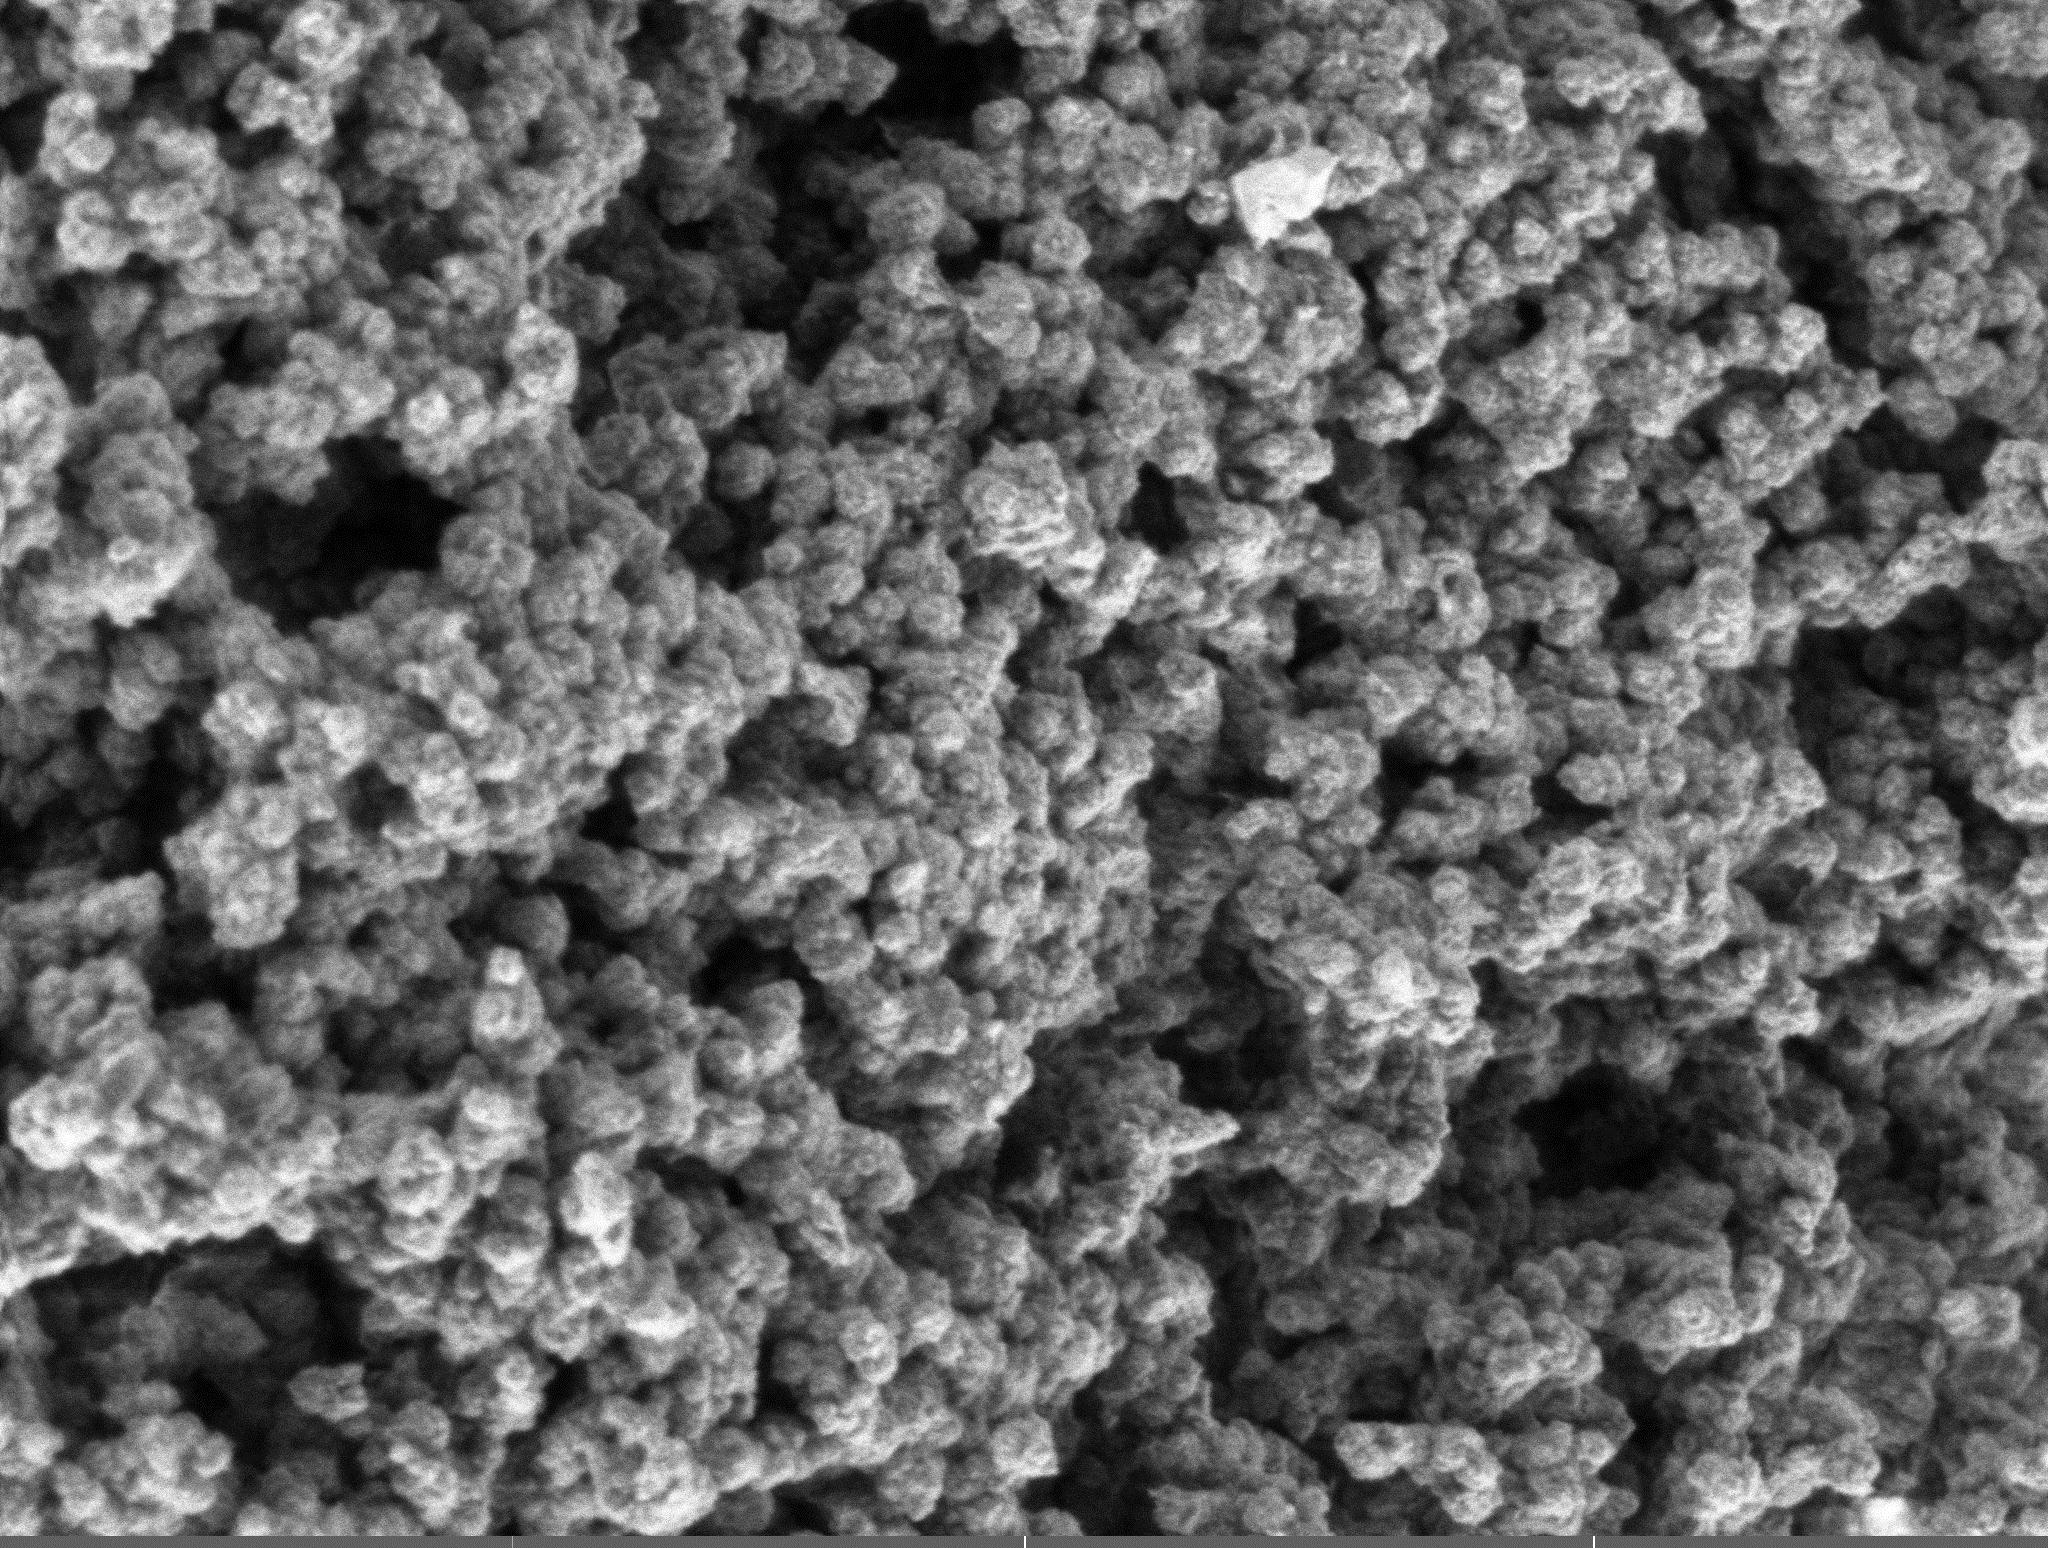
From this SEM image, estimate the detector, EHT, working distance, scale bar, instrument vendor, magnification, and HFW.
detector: SE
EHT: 1 kV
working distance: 4.95 mm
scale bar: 5000 nm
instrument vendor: Tescan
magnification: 28.1 K X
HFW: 18 µm